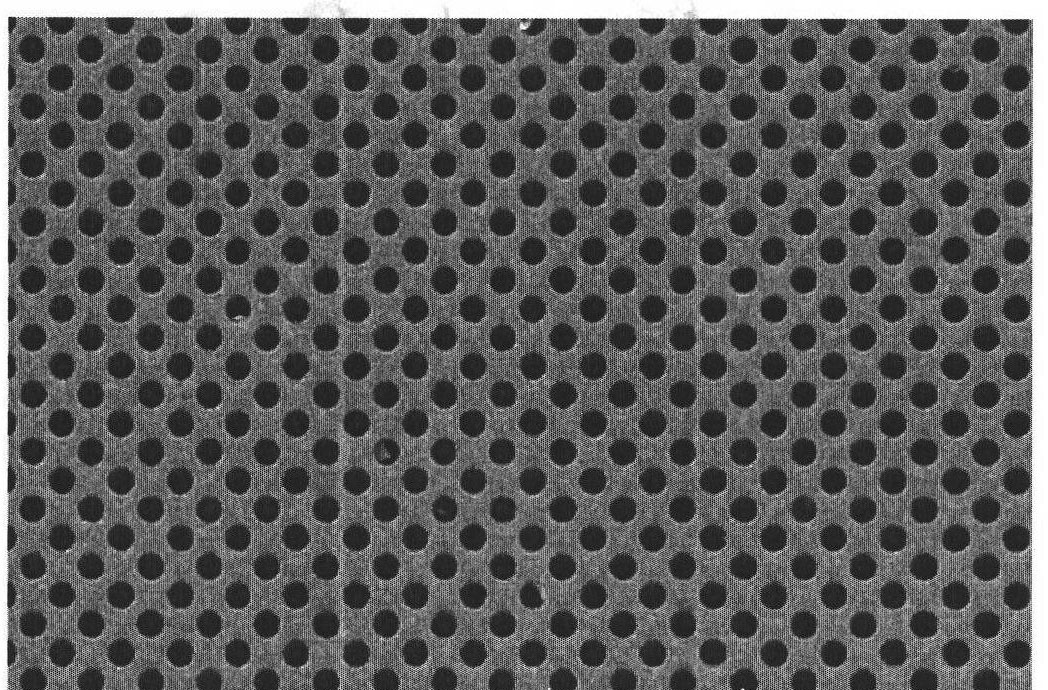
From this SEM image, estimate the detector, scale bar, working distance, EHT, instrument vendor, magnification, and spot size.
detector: SE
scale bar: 5000 nm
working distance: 7.5 mm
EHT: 5 kV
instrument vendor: FEI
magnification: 4.537 K X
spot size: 3